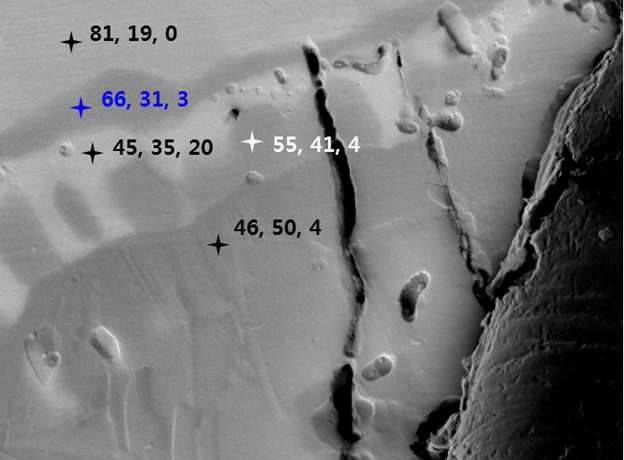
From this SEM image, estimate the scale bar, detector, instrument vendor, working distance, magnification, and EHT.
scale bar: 1000 nm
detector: BED-C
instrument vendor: JEOL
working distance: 11.5 mm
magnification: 15 K X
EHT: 15 kV